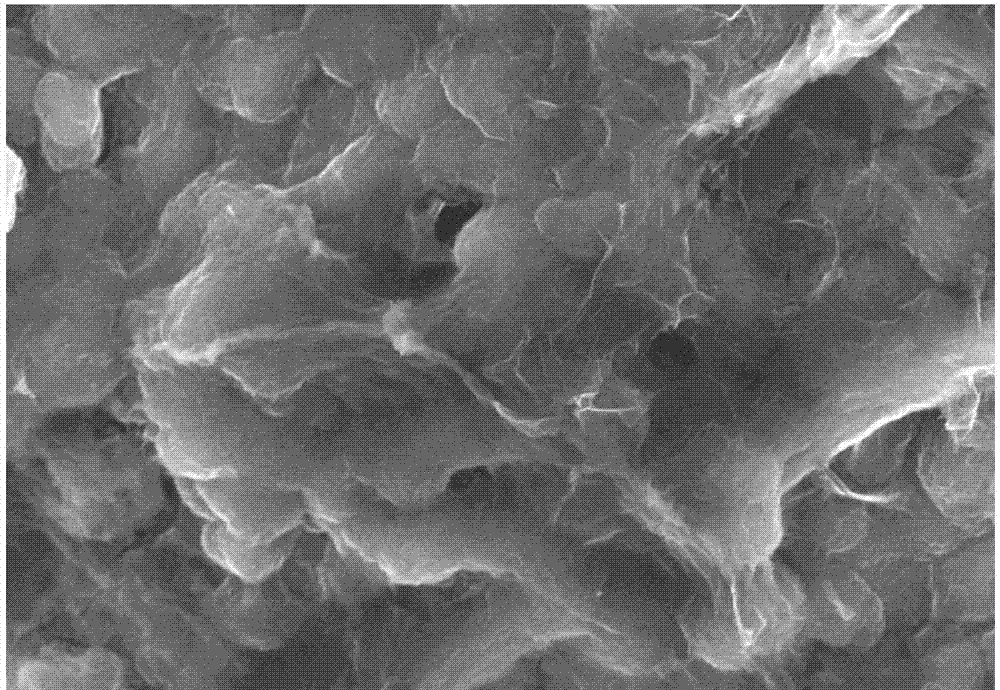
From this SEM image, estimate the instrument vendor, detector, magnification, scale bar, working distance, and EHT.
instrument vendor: Hitachi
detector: SE(U)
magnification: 10 K X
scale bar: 5000 nm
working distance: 9.3 mm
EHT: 10 kV